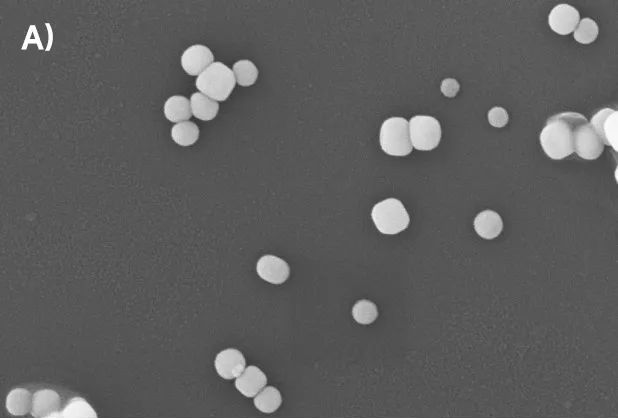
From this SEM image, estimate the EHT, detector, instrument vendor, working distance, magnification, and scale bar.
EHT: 15 kV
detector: SE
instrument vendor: other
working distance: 6.93854 mm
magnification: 50 K X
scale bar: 700 nm